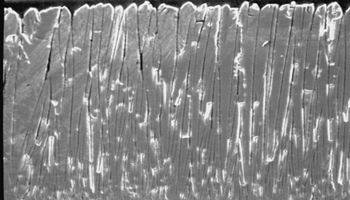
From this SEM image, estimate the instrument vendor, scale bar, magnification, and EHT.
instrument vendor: JEOL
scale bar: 25000 nm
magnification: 0.4 K X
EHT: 20 kV